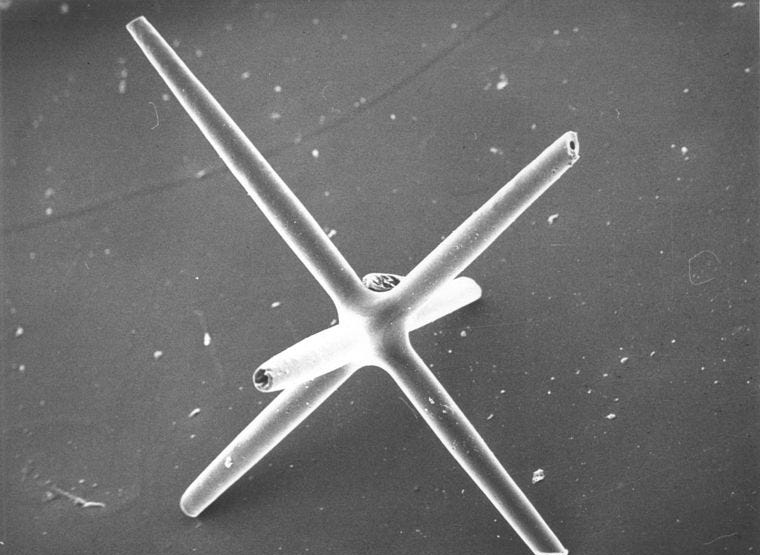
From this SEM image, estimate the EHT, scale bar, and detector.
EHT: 20 kV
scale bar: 400000 nm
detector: S